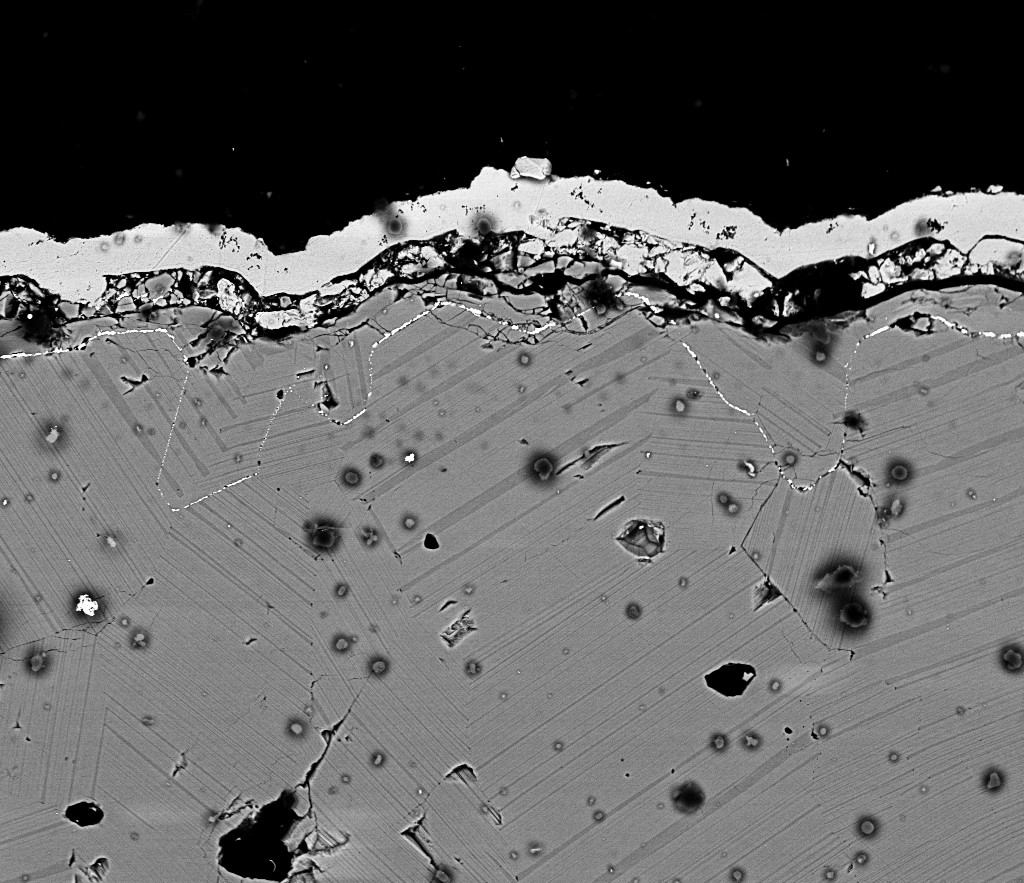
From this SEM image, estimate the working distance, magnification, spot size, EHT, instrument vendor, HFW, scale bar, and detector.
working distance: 6.6 mm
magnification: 2 K X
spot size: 4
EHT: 18 kV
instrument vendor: FEI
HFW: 149 µm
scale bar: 50000 nm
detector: BSED